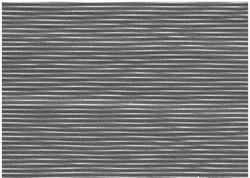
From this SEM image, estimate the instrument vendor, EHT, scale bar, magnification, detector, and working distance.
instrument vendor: JEOL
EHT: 15 kV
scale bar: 1000 nm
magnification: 10 K X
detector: BED-C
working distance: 6.5 mm